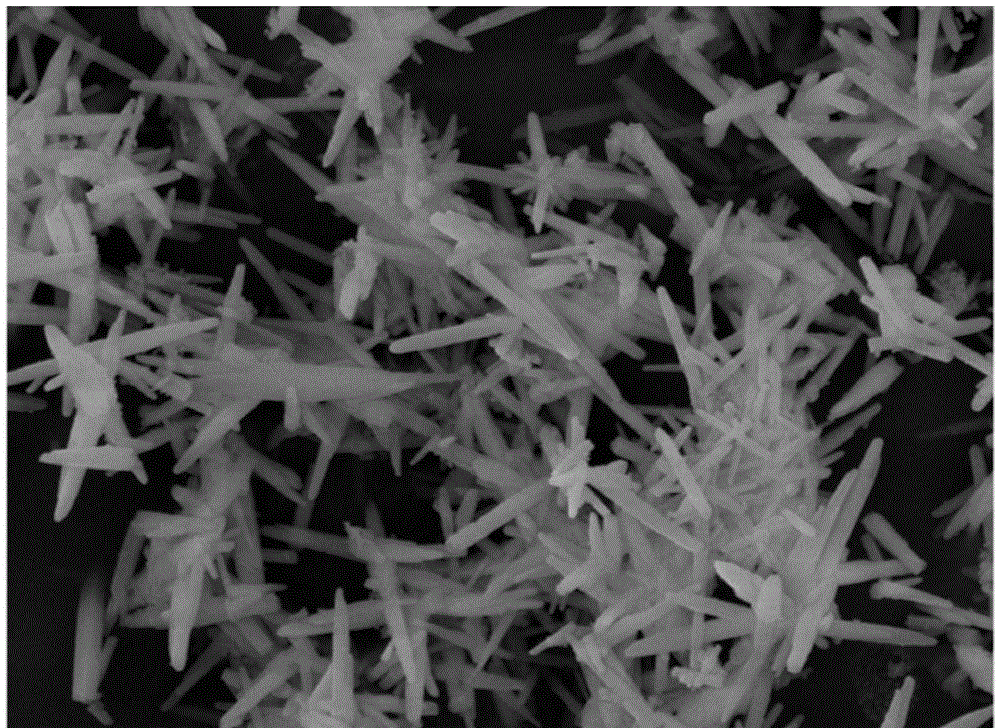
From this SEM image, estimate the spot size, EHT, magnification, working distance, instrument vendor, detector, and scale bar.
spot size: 3.5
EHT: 10 kV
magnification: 20 K X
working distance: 7.8 mm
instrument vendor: FEI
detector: ETD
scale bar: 3000 nm